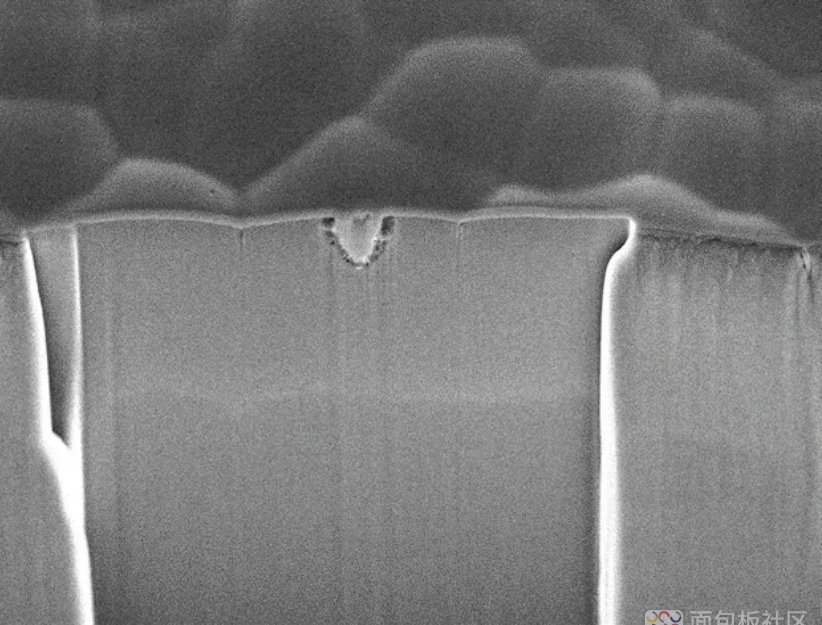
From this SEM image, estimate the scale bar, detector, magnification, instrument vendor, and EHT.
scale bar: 1000 nm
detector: SEI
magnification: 7 K X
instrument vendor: JEOL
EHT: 10 kV